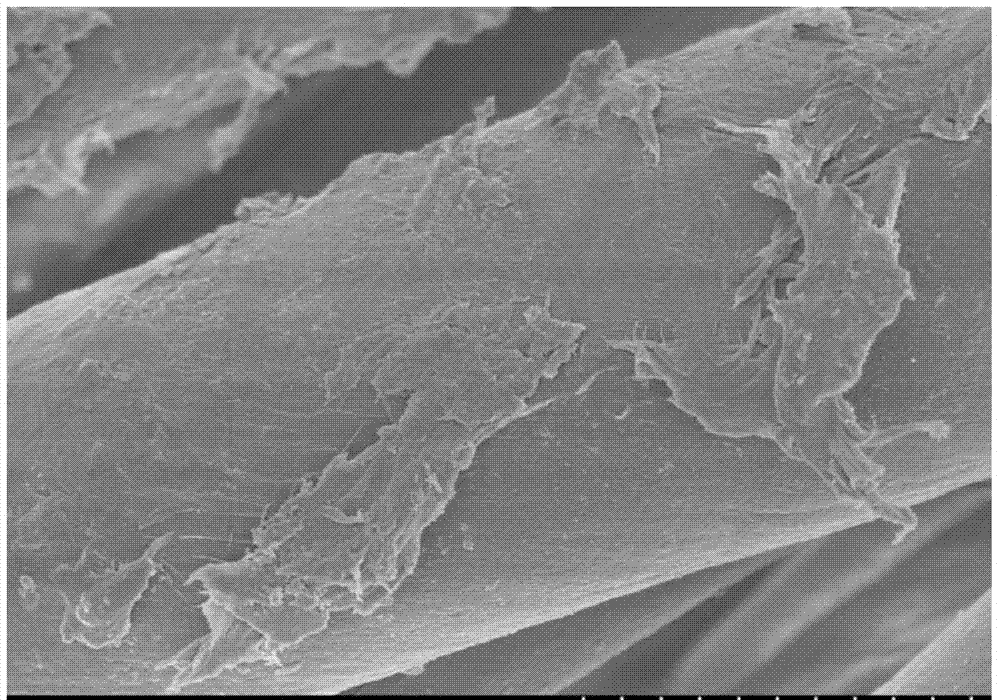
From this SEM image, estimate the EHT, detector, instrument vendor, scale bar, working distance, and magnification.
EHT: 3 kV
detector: SE(M)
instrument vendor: Hitachi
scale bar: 10000 nm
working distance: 8.4 mm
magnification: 5 K X